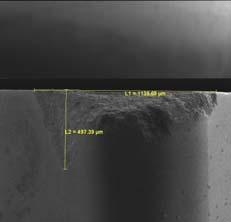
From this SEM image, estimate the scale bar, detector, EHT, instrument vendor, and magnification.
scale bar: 500000 nm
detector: SE Detector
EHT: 5 kV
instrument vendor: Tescan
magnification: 0.15 K X